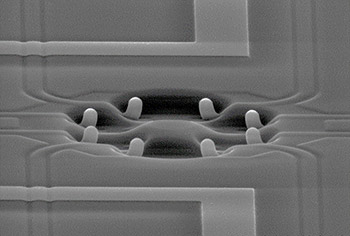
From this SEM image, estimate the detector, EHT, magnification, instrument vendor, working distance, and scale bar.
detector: SE(U)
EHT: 30 kV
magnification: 2 K X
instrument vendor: Hitachi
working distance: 21 mm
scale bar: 20000 nm